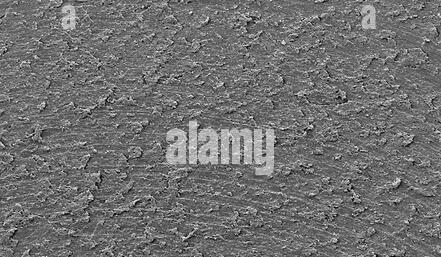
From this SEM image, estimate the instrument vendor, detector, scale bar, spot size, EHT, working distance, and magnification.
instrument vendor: FEI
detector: SE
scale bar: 200000 nm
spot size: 3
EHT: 10 kV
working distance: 19.9 mm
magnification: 0.127 K X